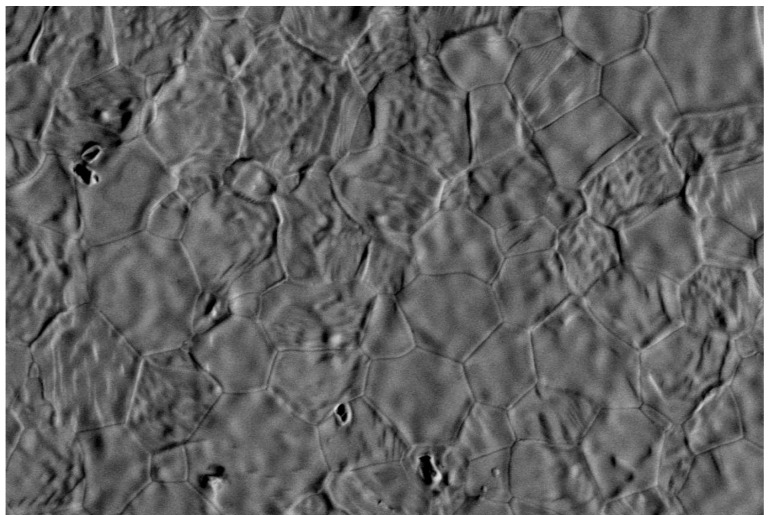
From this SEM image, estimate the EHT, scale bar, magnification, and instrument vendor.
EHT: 15 kV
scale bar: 20000 nm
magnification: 0.9 K X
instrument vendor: JEOL